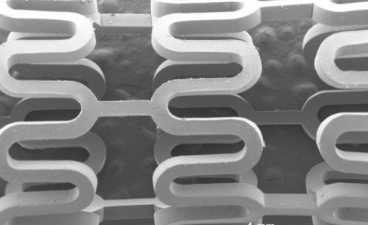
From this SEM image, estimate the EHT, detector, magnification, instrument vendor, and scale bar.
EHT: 15 kV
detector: SEI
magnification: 0.03 K X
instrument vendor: JEOL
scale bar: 1000000 nm